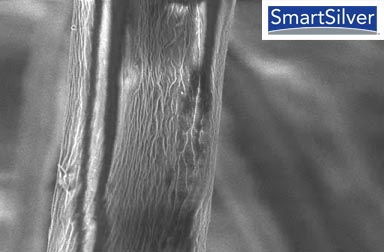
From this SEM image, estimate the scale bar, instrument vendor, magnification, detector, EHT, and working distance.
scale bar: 2000 nm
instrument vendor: Zeiss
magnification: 2.65 K X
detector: InLens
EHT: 3 kV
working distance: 4 mm